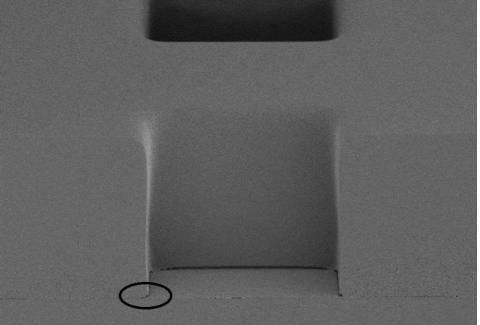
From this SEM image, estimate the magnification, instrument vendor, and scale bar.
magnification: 1 K X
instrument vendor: Hitachi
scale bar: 50000 nm